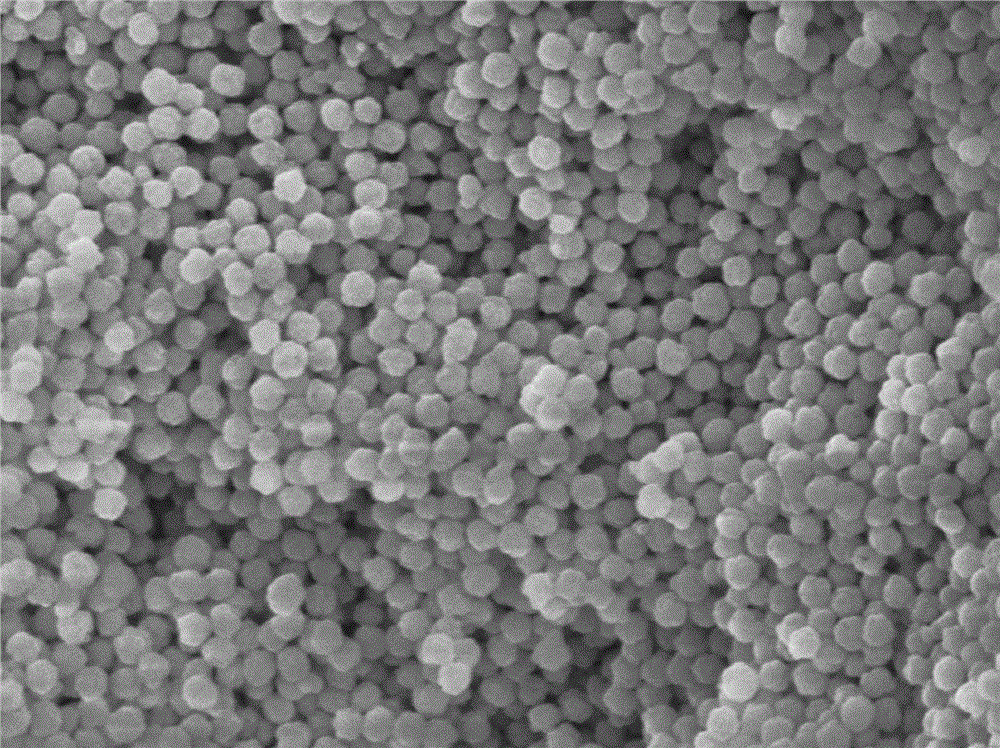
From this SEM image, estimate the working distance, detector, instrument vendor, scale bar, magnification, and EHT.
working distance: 15 mm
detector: SEI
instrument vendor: JEOL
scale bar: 1000 nm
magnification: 15 K X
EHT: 15 kV